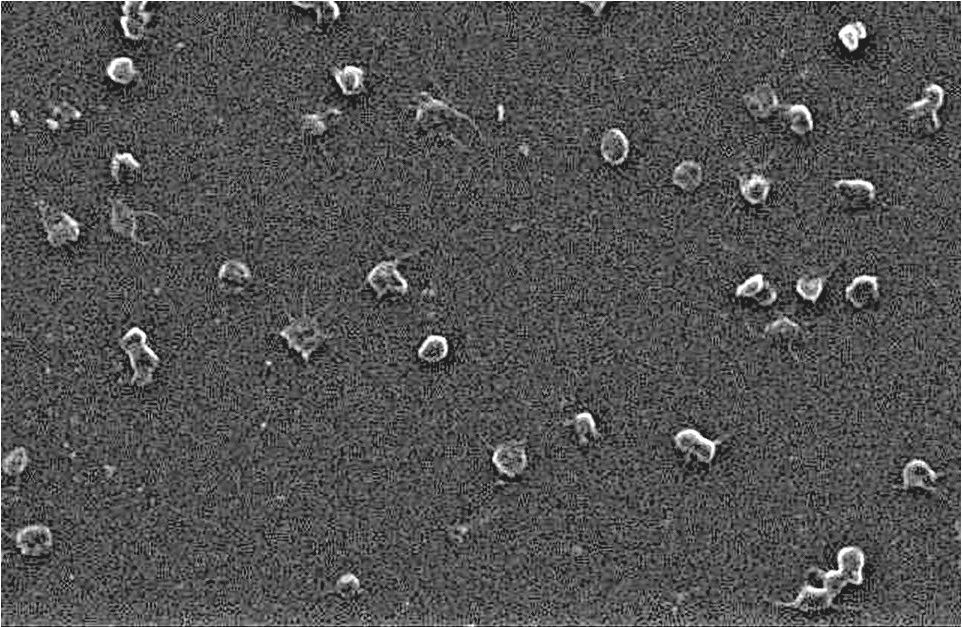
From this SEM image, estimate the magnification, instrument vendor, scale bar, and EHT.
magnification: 1.5 K X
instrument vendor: JEOL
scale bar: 10000 nm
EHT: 5 kV